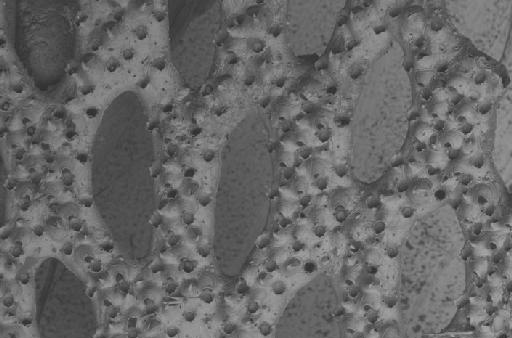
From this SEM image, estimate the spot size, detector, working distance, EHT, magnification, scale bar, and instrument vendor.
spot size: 550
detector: BSD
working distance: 16 mm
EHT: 20 kV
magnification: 0.06 K X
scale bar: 200000 nm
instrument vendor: Zeiss